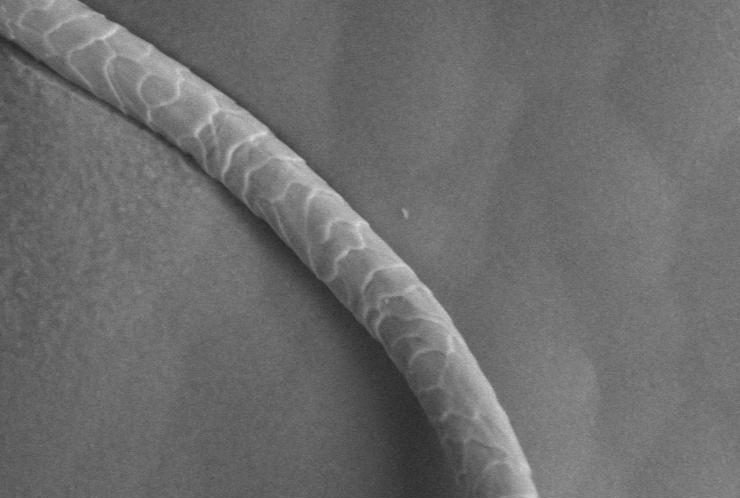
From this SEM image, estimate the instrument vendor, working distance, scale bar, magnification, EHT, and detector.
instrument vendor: JEOL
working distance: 11 mm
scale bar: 50000 nm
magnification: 0.5 K X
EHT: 15 kV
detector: BES